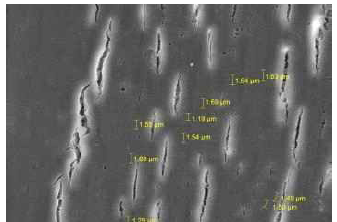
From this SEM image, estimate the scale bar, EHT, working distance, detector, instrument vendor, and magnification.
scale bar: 10000 nm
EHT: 10 kV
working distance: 10.16 mm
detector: SE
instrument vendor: Tescan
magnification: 5 K X